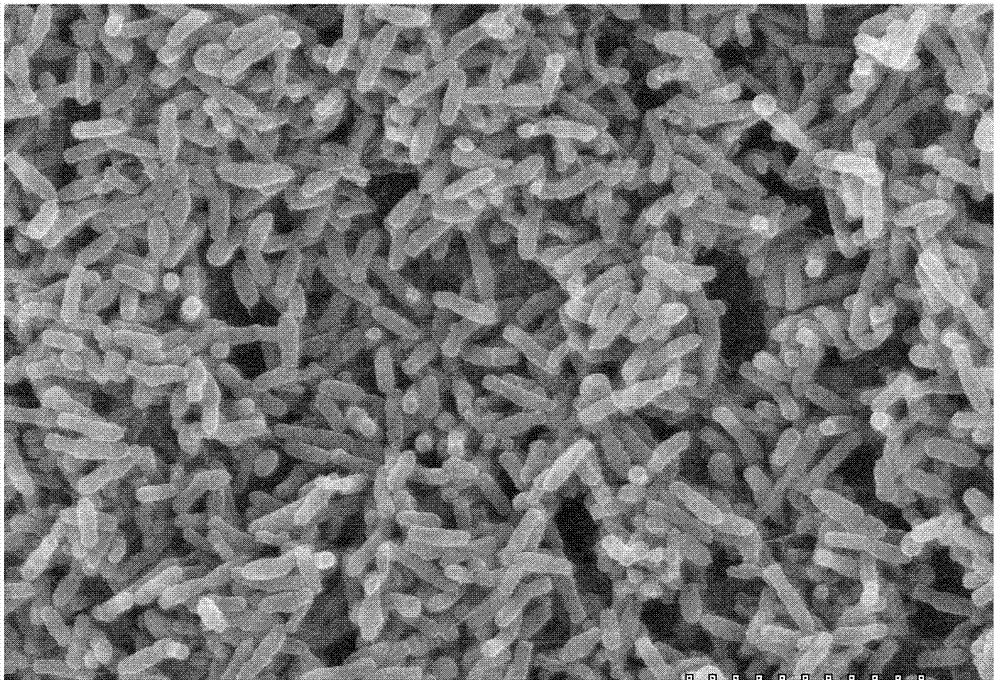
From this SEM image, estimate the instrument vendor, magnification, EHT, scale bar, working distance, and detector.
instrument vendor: JEOL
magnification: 6 K X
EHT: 25 kV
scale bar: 5000 nm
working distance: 9.2 mm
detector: SE